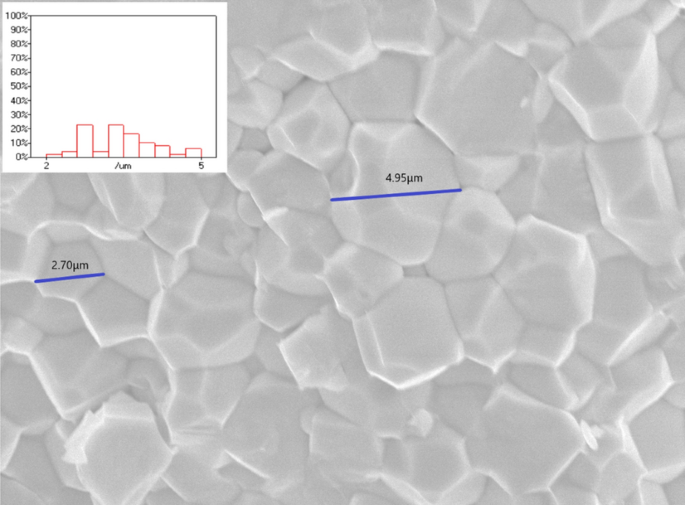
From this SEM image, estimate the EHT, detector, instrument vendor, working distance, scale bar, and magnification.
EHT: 15 kV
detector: SEI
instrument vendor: JEOL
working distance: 17 mm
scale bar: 1000 nm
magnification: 4.5 K X